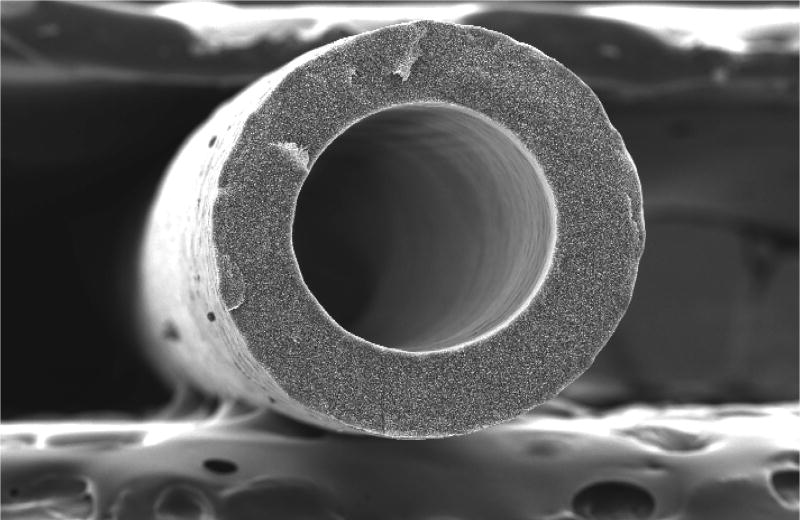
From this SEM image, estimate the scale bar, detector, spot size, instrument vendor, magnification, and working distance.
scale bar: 200000 nm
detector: SE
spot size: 3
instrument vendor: FEI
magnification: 0.2 K X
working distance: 10.1 mm